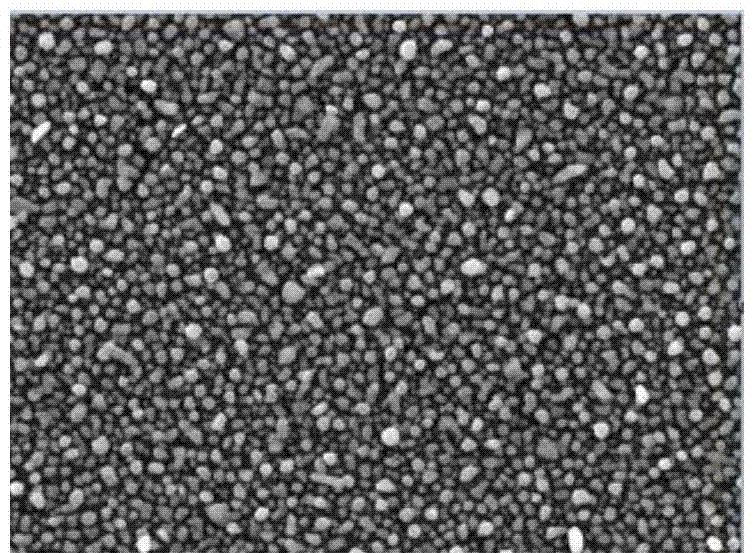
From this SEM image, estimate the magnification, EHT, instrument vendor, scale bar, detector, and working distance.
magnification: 30 K X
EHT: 10 kV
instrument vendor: JEOL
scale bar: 100 nm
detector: LED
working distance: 10.1 mm